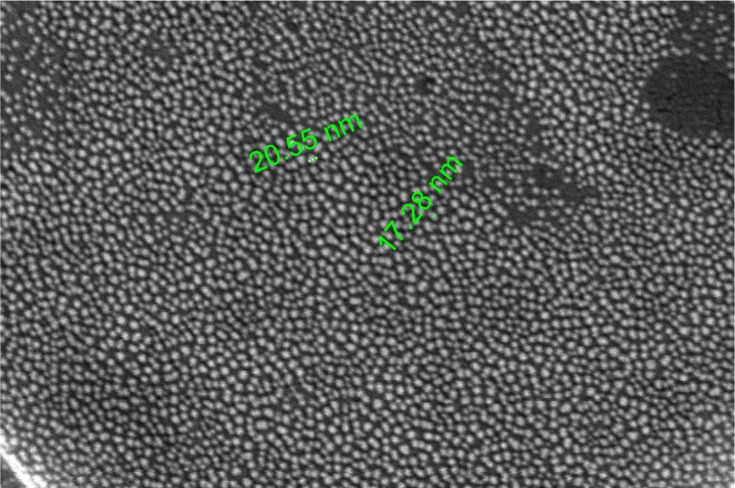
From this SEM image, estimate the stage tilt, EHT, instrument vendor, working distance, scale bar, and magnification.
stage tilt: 0°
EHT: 5 kV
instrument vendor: FEI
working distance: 4.2 mm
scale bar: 500 nm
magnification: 200 K X